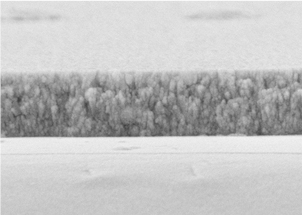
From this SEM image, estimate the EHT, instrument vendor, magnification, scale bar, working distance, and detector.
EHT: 5 kV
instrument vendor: JEOL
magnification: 50 K X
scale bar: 100 nm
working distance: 9.3 mm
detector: SEI